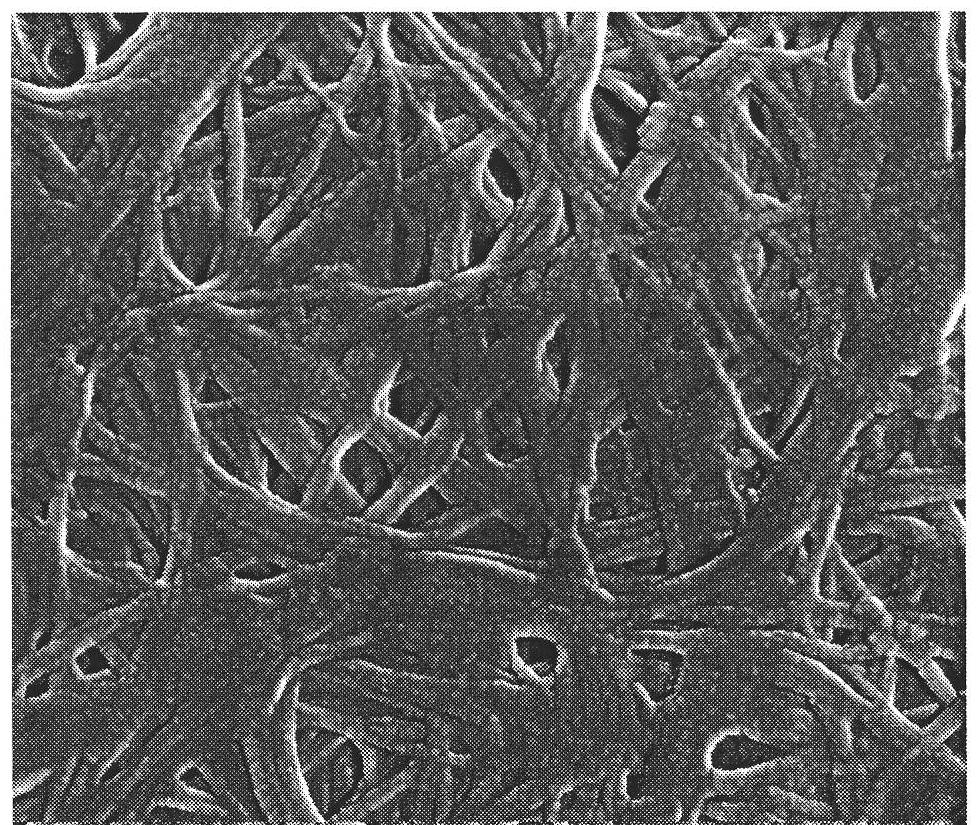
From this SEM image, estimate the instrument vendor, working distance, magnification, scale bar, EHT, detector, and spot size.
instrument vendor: FEI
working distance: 5.3 mm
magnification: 100 K X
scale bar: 500 nm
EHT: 15 kV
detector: TLD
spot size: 3